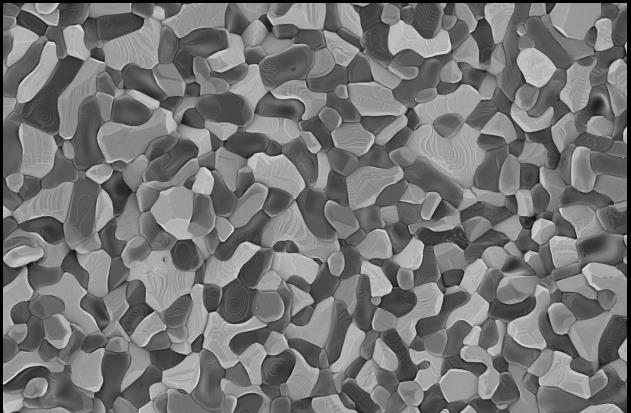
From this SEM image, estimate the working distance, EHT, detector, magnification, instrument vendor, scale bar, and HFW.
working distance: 3.6 mm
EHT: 1 kV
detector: T1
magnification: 5 K X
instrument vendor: Thermo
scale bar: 10000 nm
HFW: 25.4 µm